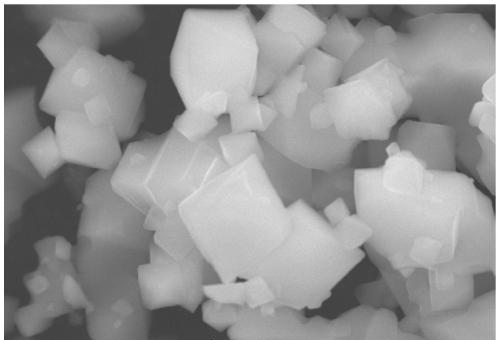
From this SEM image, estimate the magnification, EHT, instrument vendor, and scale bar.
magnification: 6 K X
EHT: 20 kV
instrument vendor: JEOL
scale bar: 2000 nm